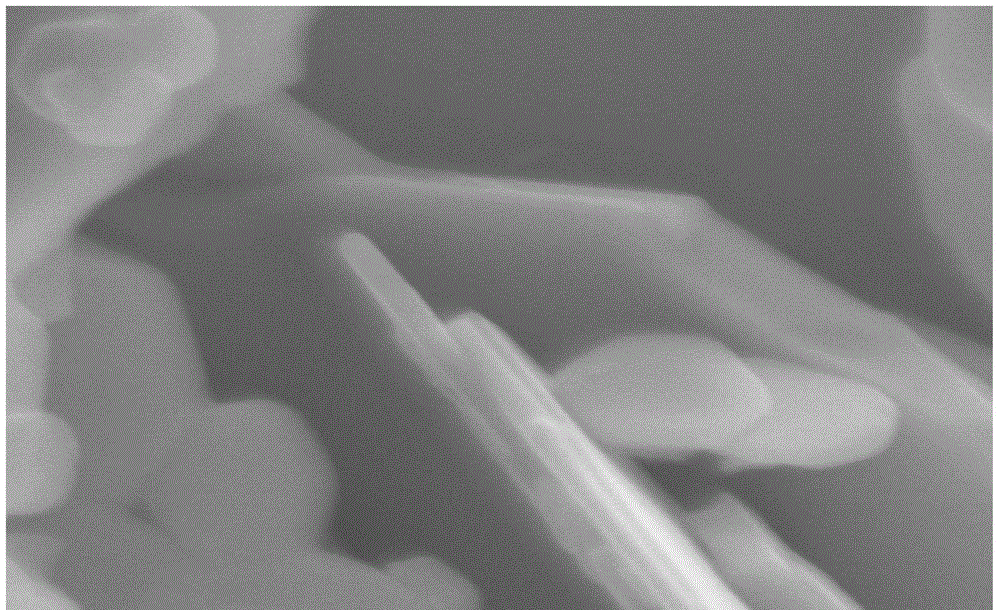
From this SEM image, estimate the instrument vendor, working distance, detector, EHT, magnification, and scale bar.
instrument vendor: Hitachi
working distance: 8.1 mm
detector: SE(M)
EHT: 5 kV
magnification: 100 K X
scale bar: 500 nm